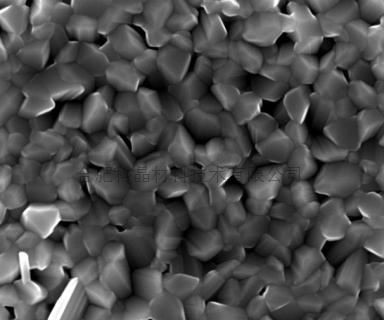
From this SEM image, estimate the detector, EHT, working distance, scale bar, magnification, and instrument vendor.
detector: TLD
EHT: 20 kV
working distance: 6 mm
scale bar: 2000 nm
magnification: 20 K X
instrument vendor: FEI